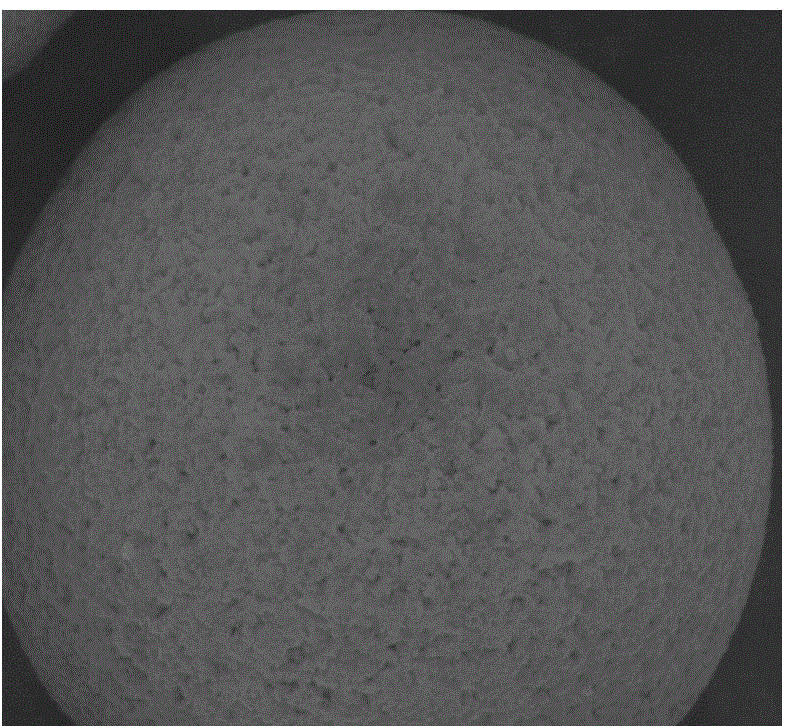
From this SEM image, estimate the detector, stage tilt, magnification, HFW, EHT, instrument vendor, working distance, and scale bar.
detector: BSED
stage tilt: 0°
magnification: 10 K X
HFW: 29.8 µm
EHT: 15 kV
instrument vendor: FEI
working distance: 11 mm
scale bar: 10000 nm